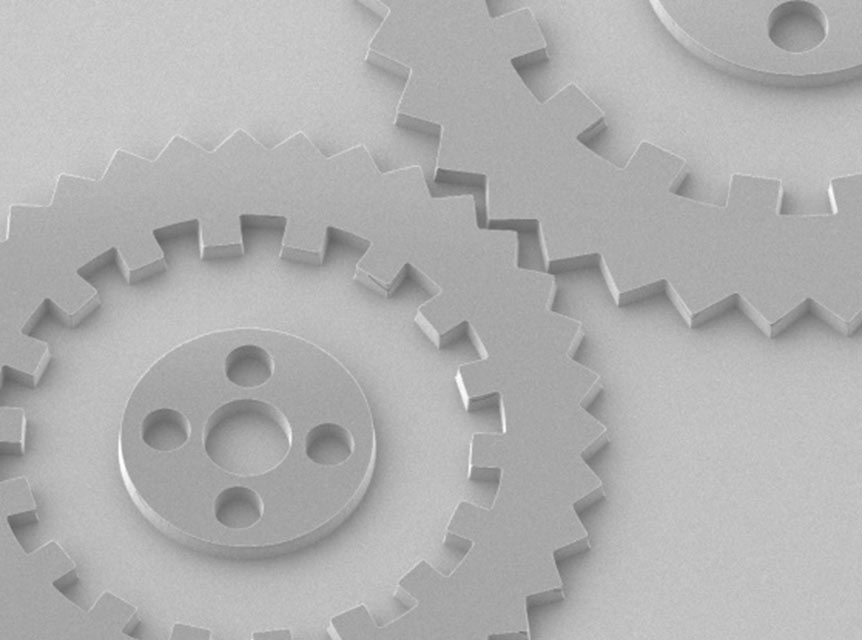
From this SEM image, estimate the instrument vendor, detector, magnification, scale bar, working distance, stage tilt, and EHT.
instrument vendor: JEOL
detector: SEI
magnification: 0.15 K X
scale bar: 100000 nm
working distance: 8 mm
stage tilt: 31°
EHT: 2 kV